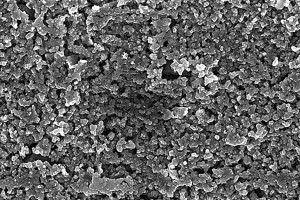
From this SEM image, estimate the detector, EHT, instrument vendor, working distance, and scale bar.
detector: InLens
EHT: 6 kV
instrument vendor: Zeiss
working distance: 6.2 mm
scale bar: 1000 nm